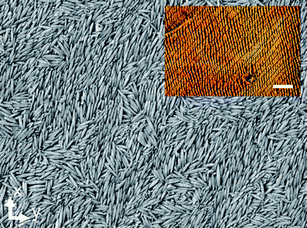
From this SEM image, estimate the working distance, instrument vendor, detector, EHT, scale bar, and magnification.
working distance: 8 mm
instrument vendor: JEOL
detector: LEI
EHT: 3 kV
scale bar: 1000 nm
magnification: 25 K X